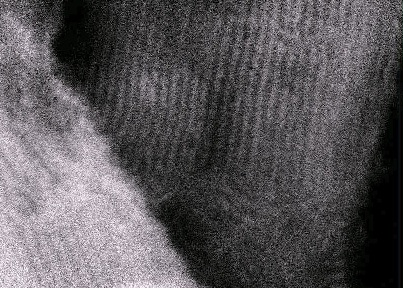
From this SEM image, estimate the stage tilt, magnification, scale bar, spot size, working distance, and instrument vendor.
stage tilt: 0°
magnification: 256.383 K X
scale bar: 200 nm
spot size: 3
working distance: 12 mm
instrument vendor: FEI